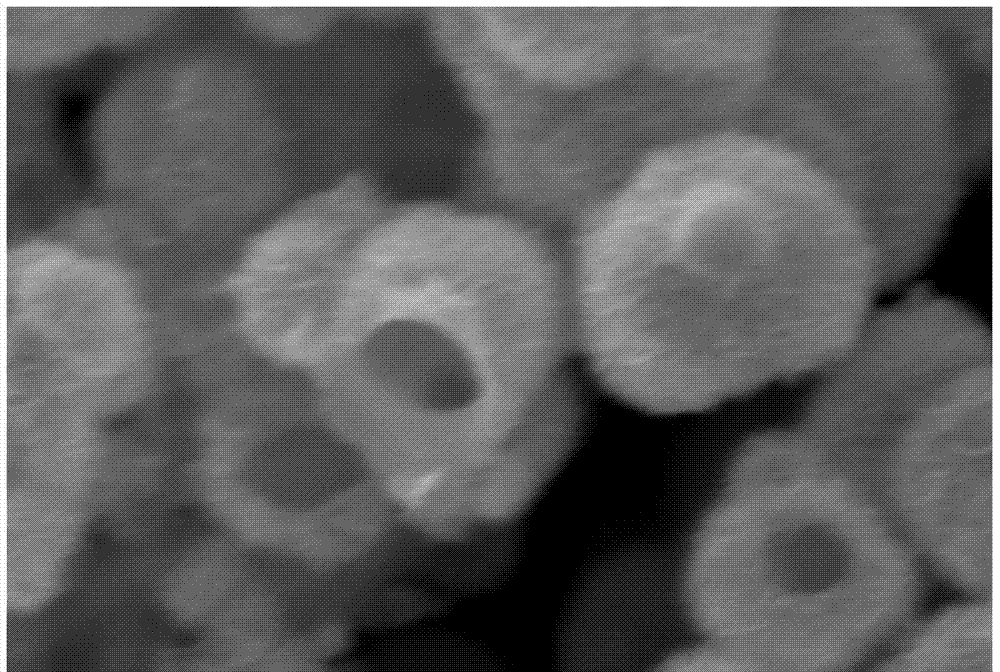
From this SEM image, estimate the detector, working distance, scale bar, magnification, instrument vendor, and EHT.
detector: SEI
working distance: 10 mm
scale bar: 1000 nm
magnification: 20 K X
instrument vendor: JEOL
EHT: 15 kV